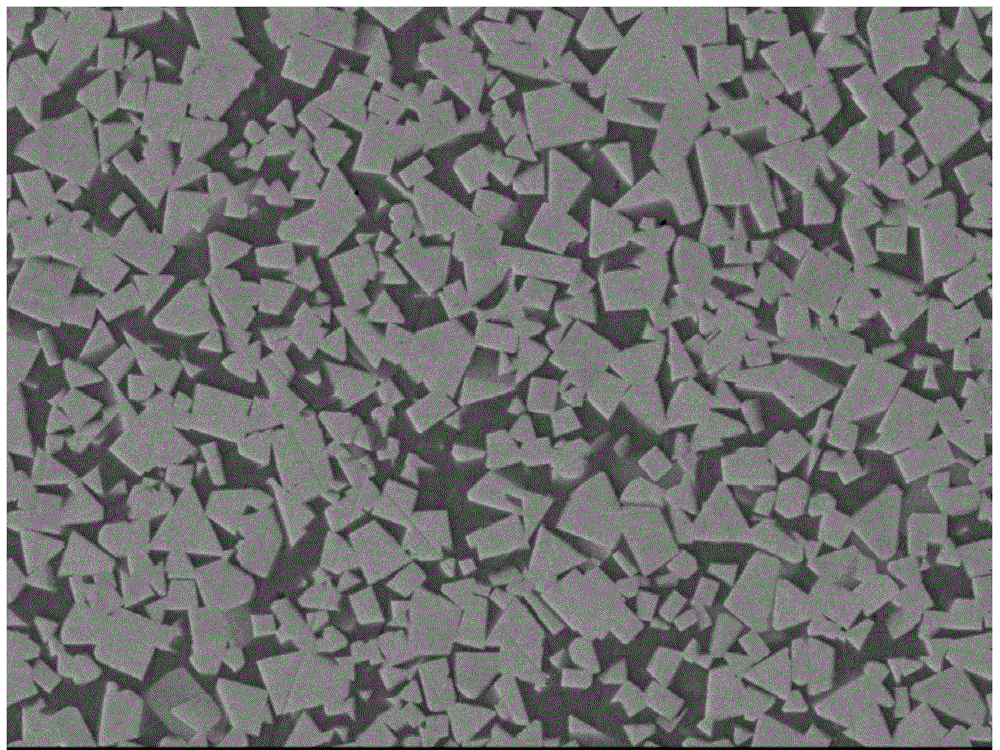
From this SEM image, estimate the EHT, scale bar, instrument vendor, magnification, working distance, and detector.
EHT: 15 kV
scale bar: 1000 nm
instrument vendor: JEOL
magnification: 3 K X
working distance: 8.7 mm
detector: LEI